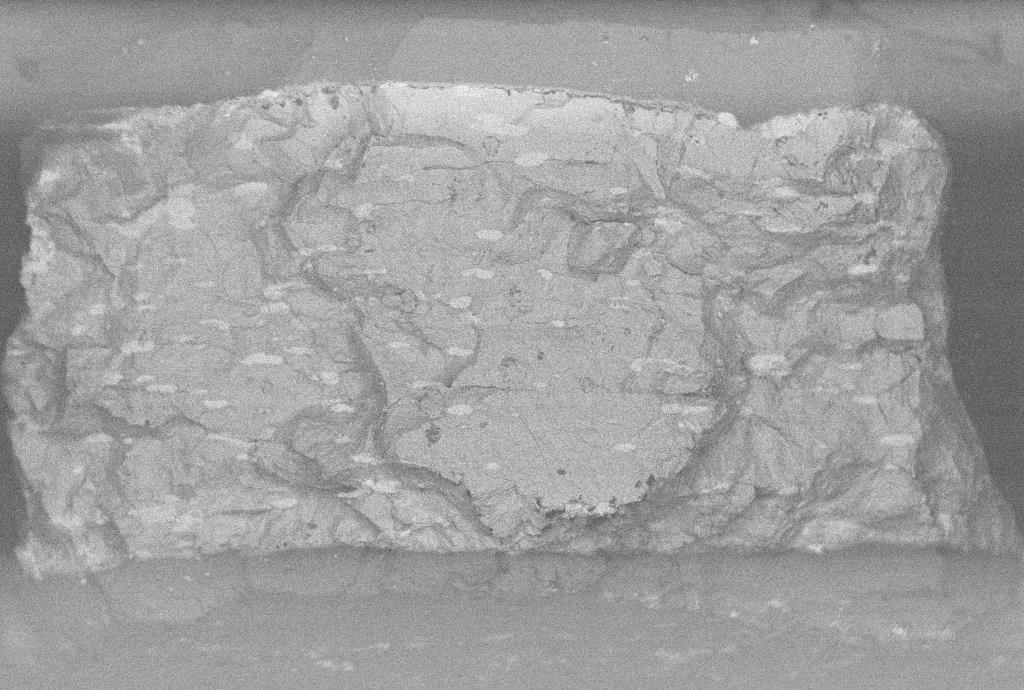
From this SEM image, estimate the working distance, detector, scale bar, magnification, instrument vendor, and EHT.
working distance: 9.5 mm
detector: QBSD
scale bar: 200000 nm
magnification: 0.091 K X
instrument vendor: Zeiss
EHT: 20 kV